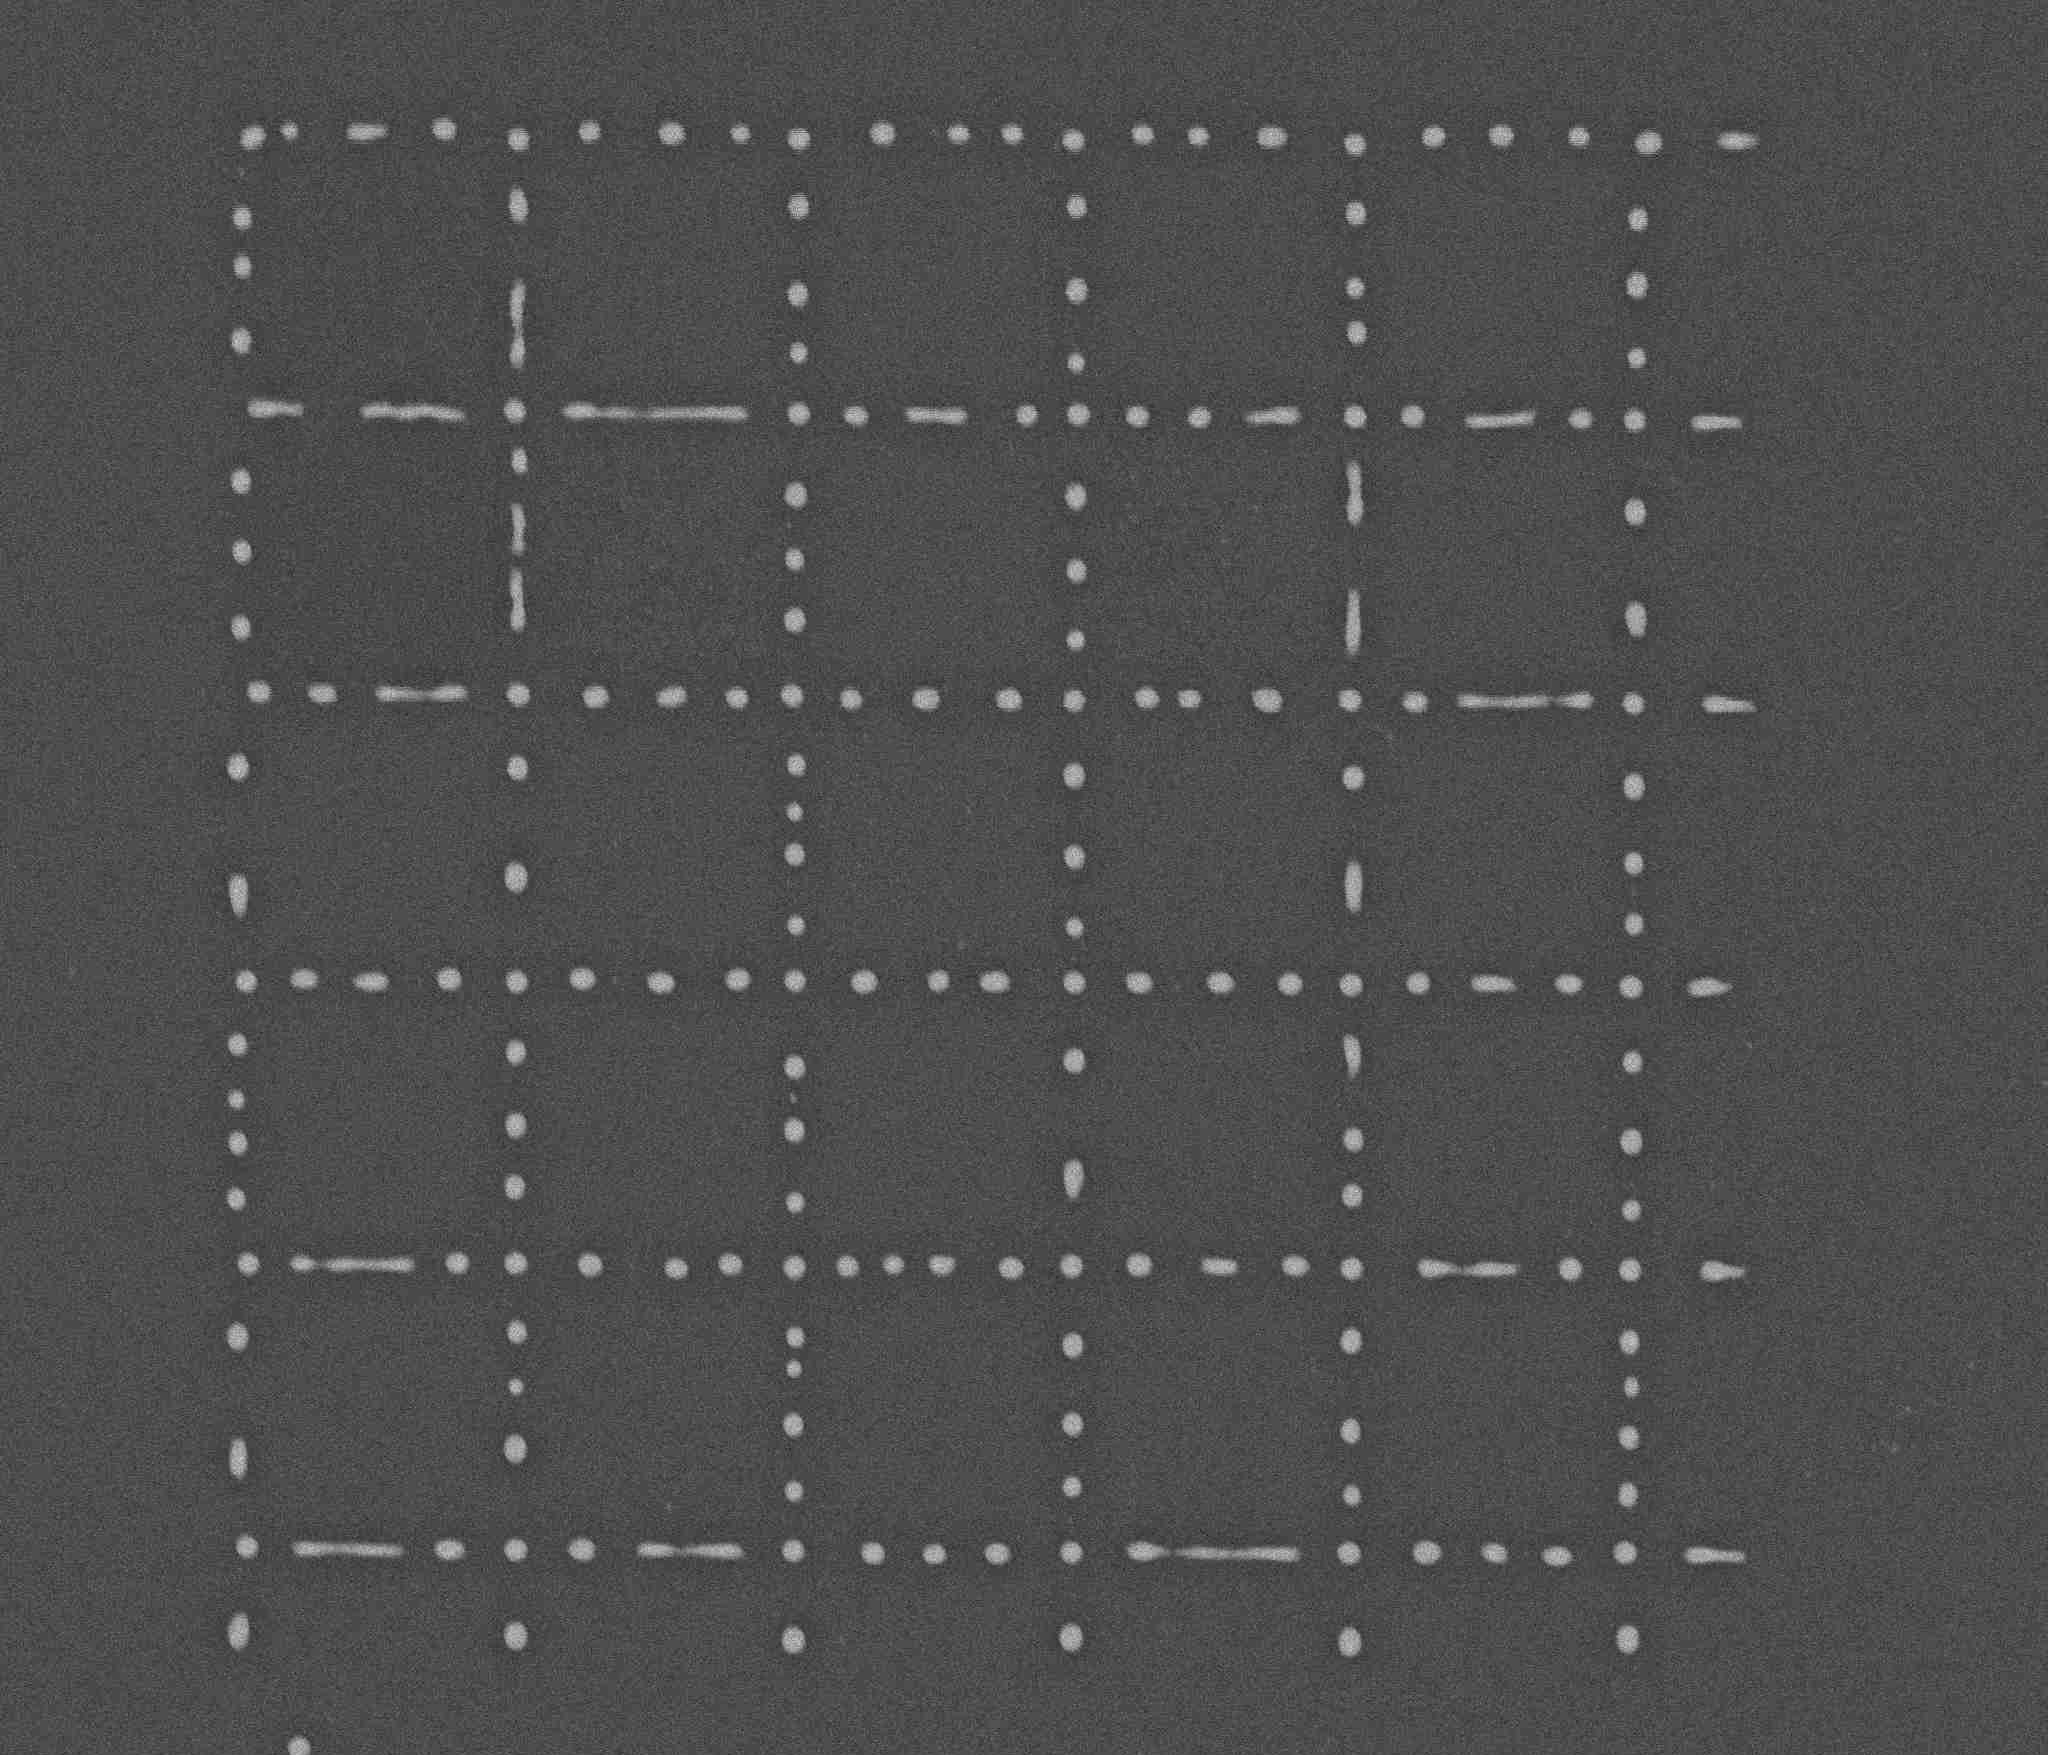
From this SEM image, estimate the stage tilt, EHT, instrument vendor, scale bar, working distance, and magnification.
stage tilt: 0°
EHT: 5 kV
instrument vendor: FEI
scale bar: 5000 nm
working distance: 5.2 mm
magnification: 10 K X